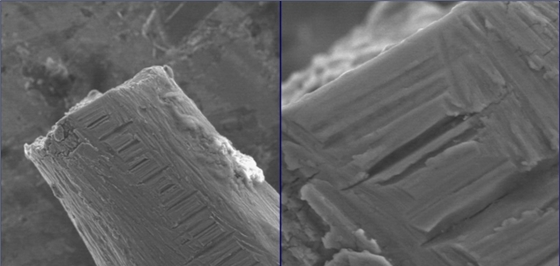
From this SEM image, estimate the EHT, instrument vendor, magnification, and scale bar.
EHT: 25 kV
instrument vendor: JEOL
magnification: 0.4 K X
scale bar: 75000 nm